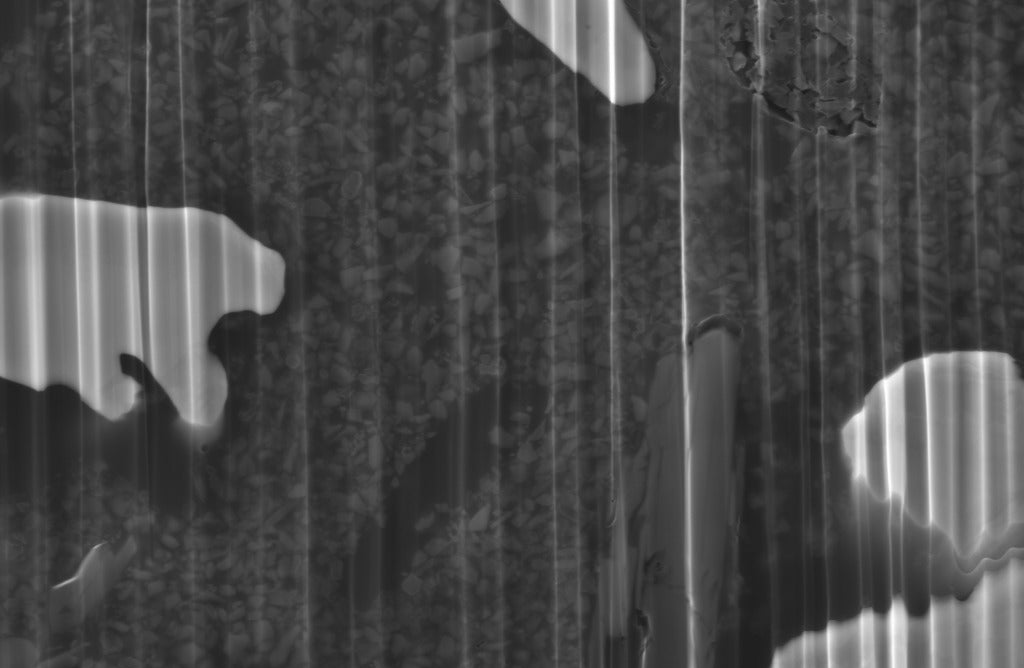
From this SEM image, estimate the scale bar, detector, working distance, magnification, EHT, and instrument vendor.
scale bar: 1000 nm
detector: SE2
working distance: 10.1 mm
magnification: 10 K X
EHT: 10 kV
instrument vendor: Zeiss